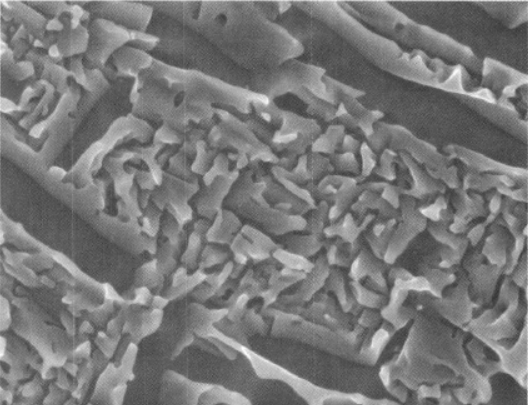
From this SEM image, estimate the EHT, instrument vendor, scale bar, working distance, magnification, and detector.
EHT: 5 kV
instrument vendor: JEOL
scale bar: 100 nm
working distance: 8 mm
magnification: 50 K X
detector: SEI